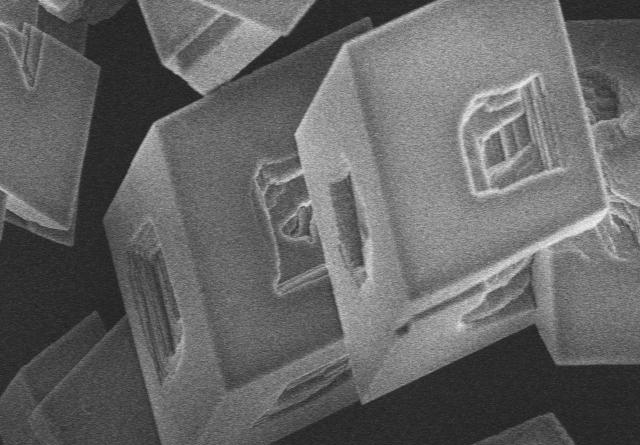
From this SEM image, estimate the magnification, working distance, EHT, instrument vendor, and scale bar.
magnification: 7 K X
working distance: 9 mm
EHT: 5 kV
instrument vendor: Hitachi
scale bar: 5000 nm